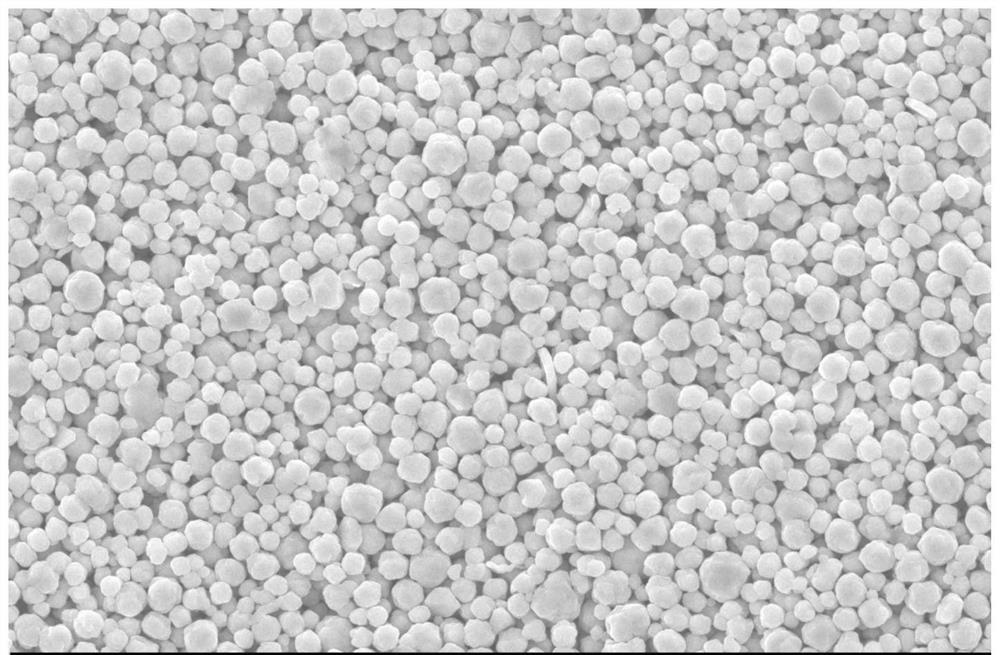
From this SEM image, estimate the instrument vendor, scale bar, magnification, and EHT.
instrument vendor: other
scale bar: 10000 nm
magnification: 2 K X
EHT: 25 kV